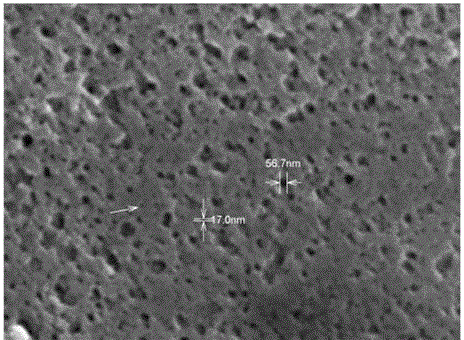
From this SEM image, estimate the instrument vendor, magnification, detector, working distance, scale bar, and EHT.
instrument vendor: Hitachi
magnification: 35 K X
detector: SE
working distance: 4.1 mm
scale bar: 1000 nm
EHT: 15 kV